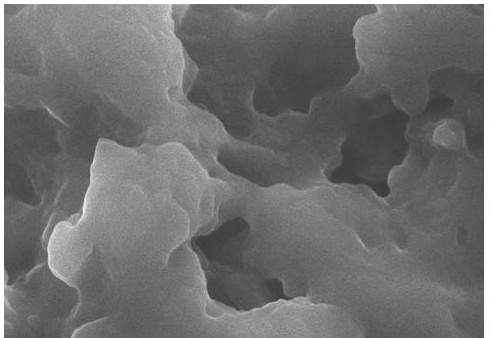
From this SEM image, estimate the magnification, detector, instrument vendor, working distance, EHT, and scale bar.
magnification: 10 K X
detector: SE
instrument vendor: Hitachi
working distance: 4.4 mm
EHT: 15 kV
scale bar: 5000 nm